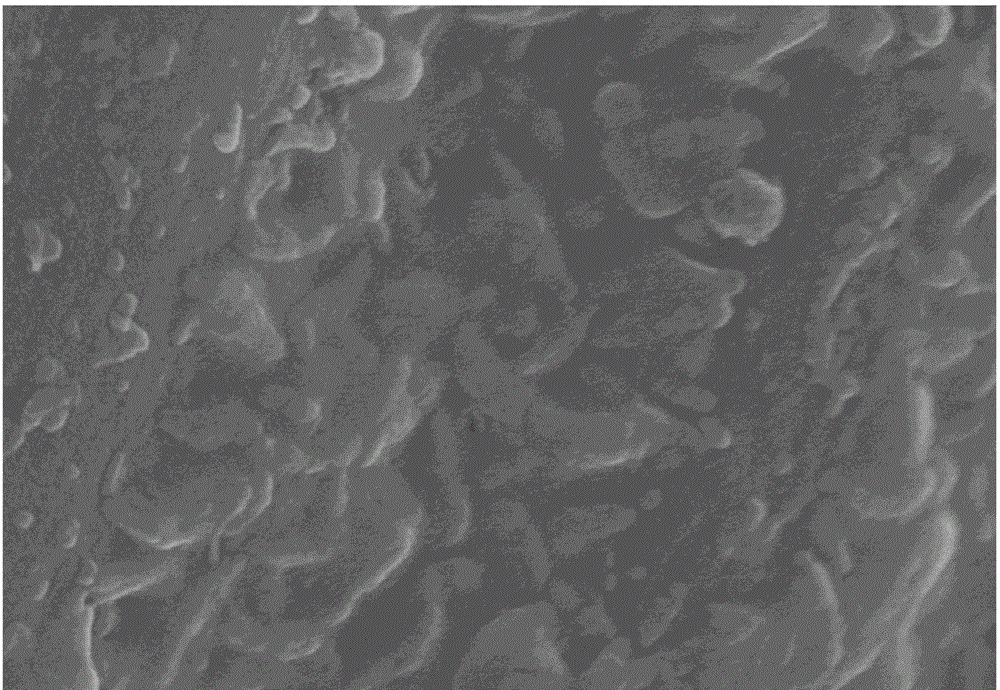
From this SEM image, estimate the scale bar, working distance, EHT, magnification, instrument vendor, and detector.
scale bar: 500 nm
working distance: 4.8 mm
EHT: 5 kV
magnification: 60 K X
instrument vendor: Hitachi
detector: SE(U)